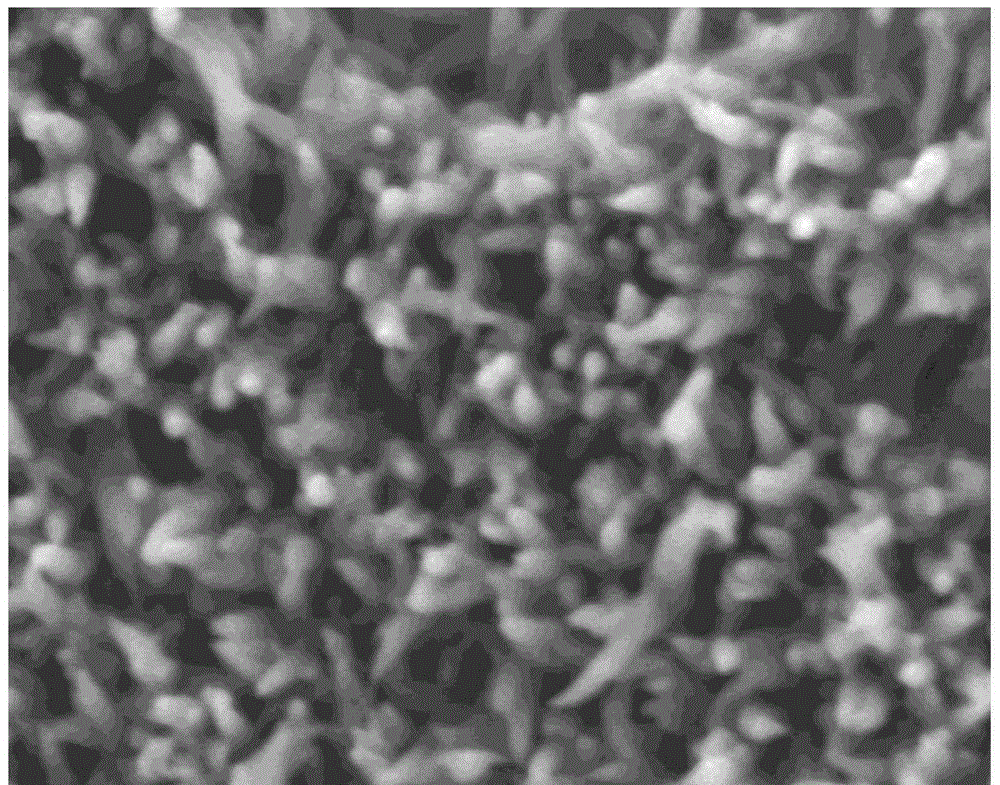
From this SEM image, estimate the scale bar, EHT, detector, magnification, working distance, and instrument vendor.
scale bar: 100 nm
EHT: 3 kV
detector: LED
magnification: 50 K X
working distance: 8 mm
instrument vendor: JEOL